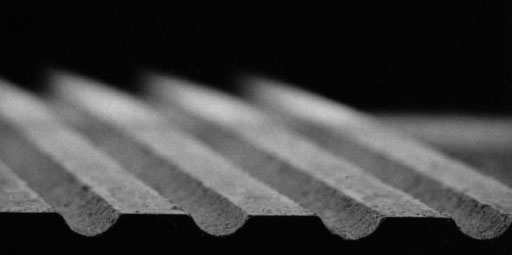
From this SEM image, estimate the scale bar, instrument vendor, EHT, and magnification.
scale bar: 100000 nm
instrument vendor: JEOL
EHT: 30 kV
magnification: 0.1 K X